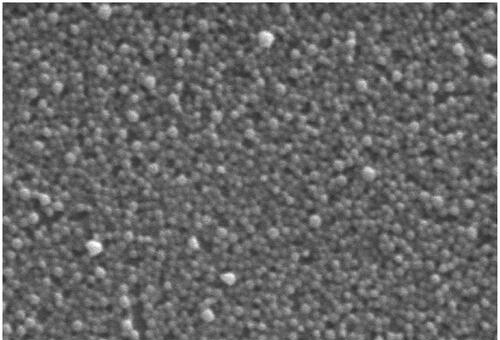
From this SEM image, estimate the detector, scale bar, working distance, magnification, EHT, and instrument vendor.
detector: SE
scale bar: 1000 nm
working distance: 4.4 mm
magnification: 30 K X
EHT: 3 kV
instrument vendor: Hitachi